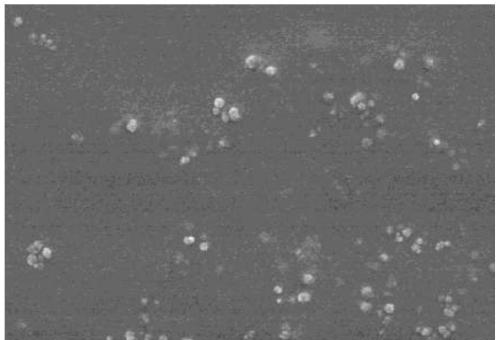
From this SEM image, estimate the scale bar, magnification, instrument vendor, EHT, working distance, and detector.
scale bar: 1000 nm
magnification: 30 K X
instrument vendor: Hitachi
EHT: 6 kV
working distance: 12.1 mm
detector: SE(U)